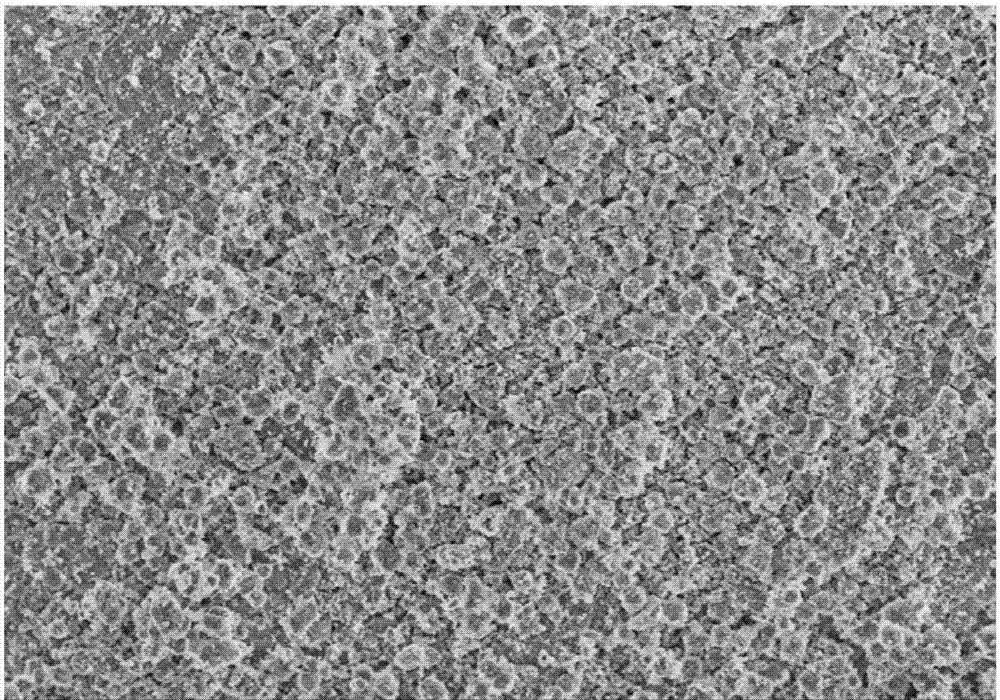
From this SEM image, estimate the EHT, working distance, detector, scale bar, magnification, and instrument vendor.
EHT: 5 kV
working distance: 8.7 mm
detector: SE(M)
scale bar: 50000 nm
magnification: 1 K X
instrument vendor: Hitachi